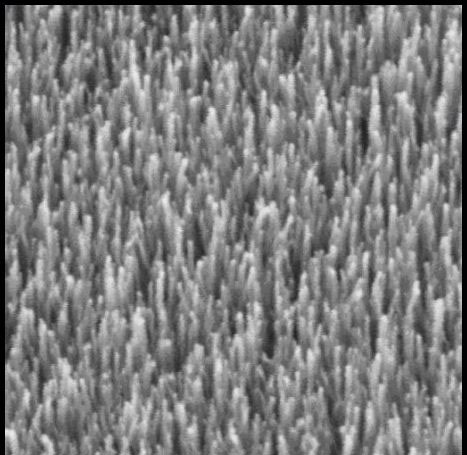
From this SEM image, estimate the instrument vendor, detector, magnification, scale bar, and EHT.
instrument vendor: Tescan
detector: SE Detector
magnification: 41.54 K X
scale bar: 1000 nm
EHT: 10 kV